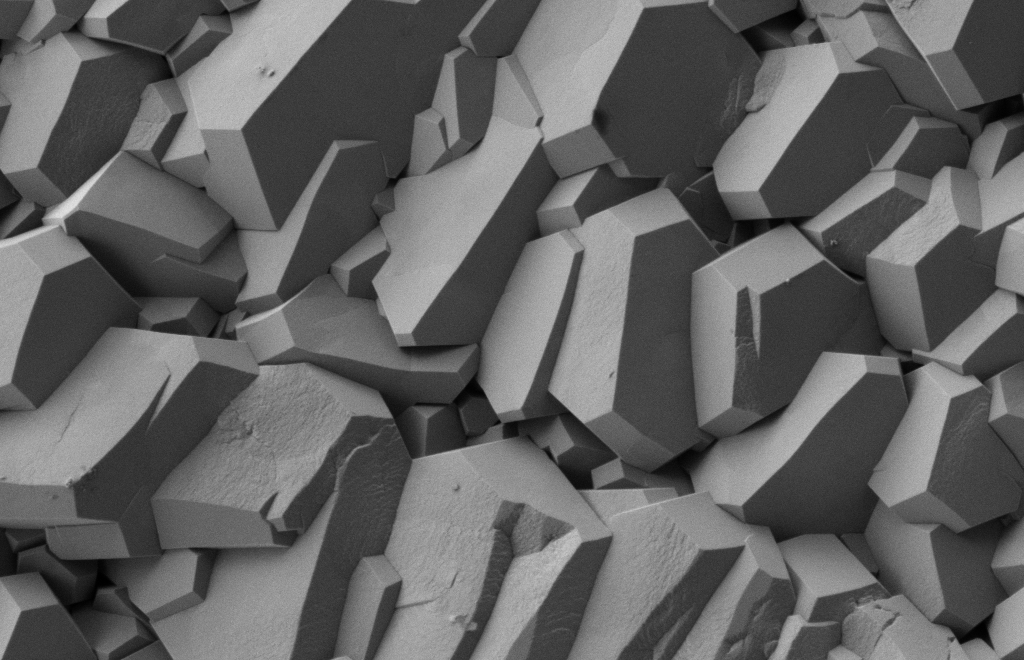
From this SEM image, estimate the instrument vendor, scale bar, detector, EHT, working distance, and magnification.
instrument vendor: Zeiss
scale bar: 1000 nm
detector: SE2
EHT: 1 kV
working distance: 4 mm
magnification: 10 K X